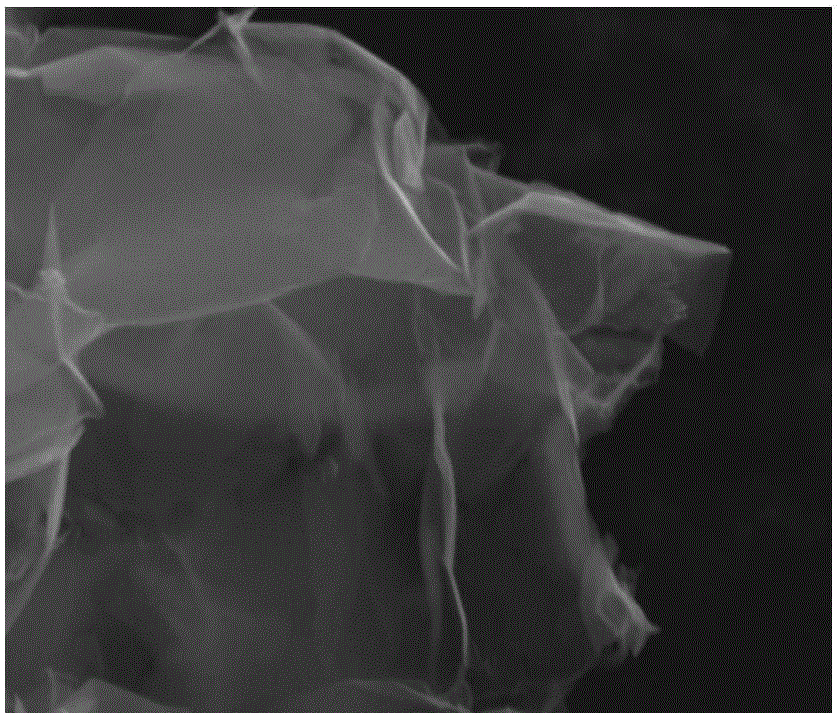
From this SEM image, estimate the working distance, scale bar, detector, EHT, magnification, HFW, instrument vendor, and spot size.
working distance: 10 mm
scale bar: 2000 nm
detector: ETD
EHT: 10 kV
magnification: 50 K X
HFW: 5.97 µm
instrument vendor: FEI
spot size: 3